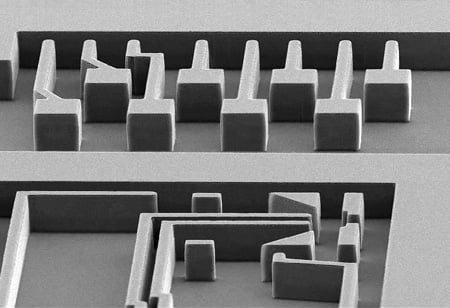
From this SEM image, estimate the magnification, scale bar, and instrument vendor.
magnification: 0.19 K X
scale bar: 100000 nm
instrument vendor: other